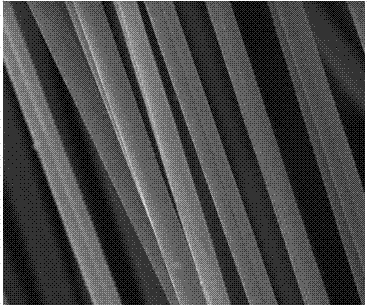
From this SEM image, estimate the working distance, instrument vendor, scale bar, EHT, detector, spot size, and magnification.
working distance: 9.8 mm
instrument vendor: FEI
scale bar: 40000 nm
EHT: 25 kV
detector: ETD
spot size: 2.5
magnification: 3 K X